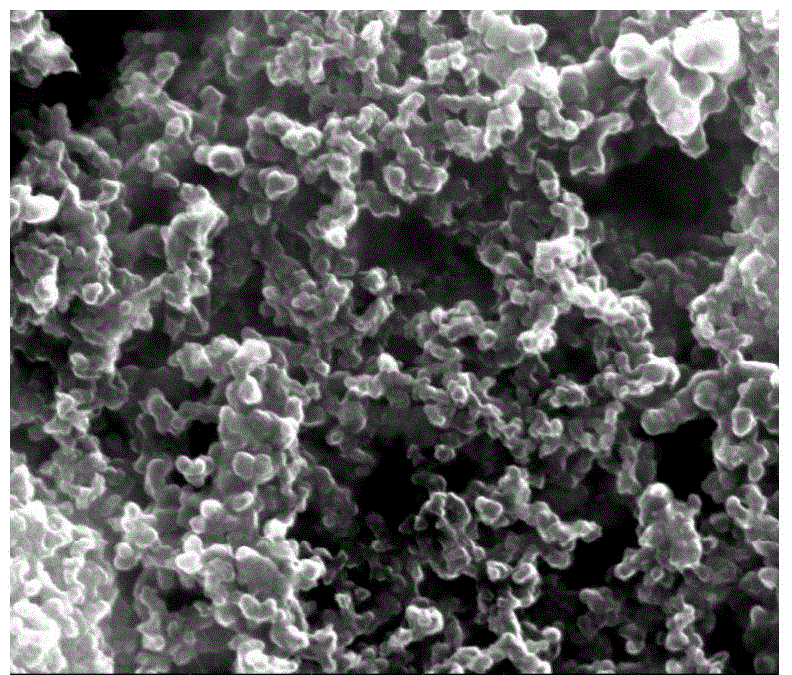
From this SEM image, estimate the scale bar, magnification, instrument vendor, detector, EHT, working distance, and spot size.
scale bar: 500 nm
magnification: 100 K X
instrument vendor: FEI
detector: ETD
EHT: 20 kV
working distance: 10 mm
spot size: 3